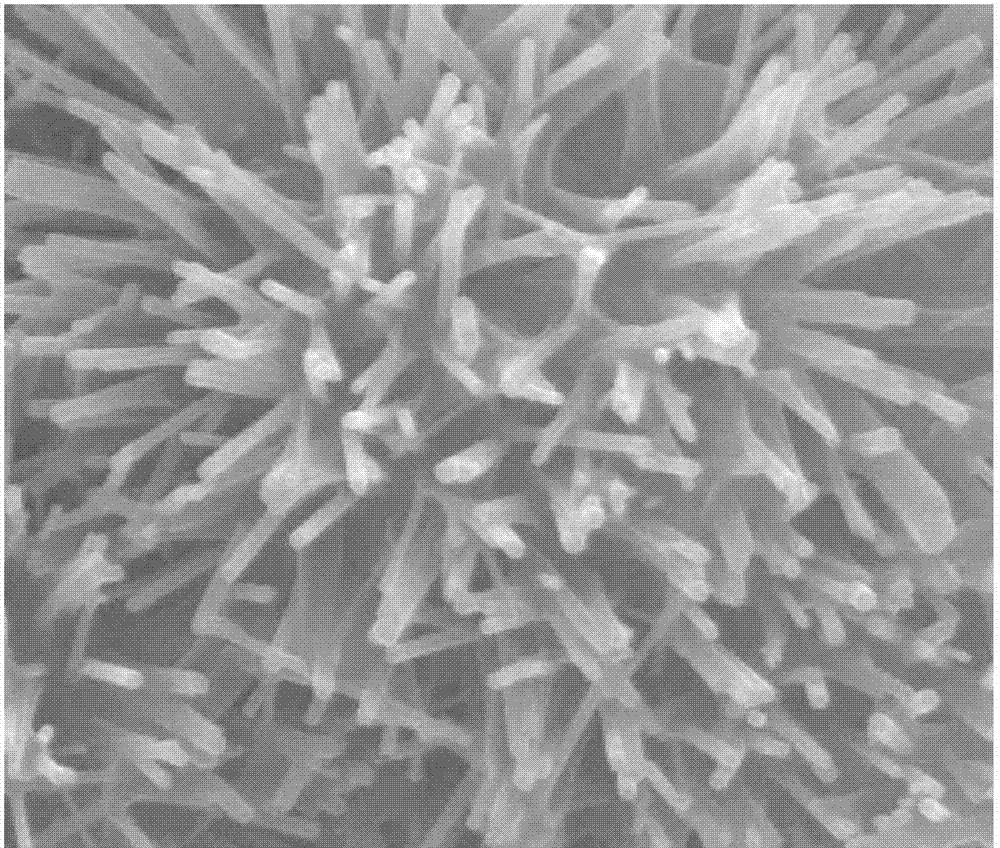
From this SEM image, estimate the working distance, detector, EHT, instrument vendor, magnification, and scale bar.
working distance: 11.9 mm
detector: SE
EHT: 30 kV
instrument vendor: FEI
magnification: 50 K X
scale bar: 2000 nm